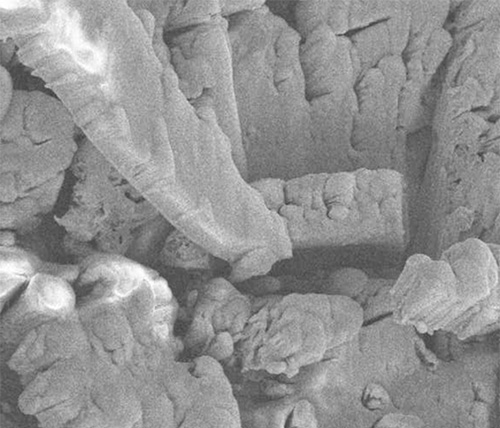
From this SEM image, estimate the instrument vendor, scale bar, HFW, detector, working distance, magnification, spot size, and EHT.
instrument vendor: FEI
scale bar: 10000 nm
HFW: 60.8 µm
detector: SED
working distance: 4.929 mm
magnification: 5 K X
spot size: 3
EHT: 10 kV